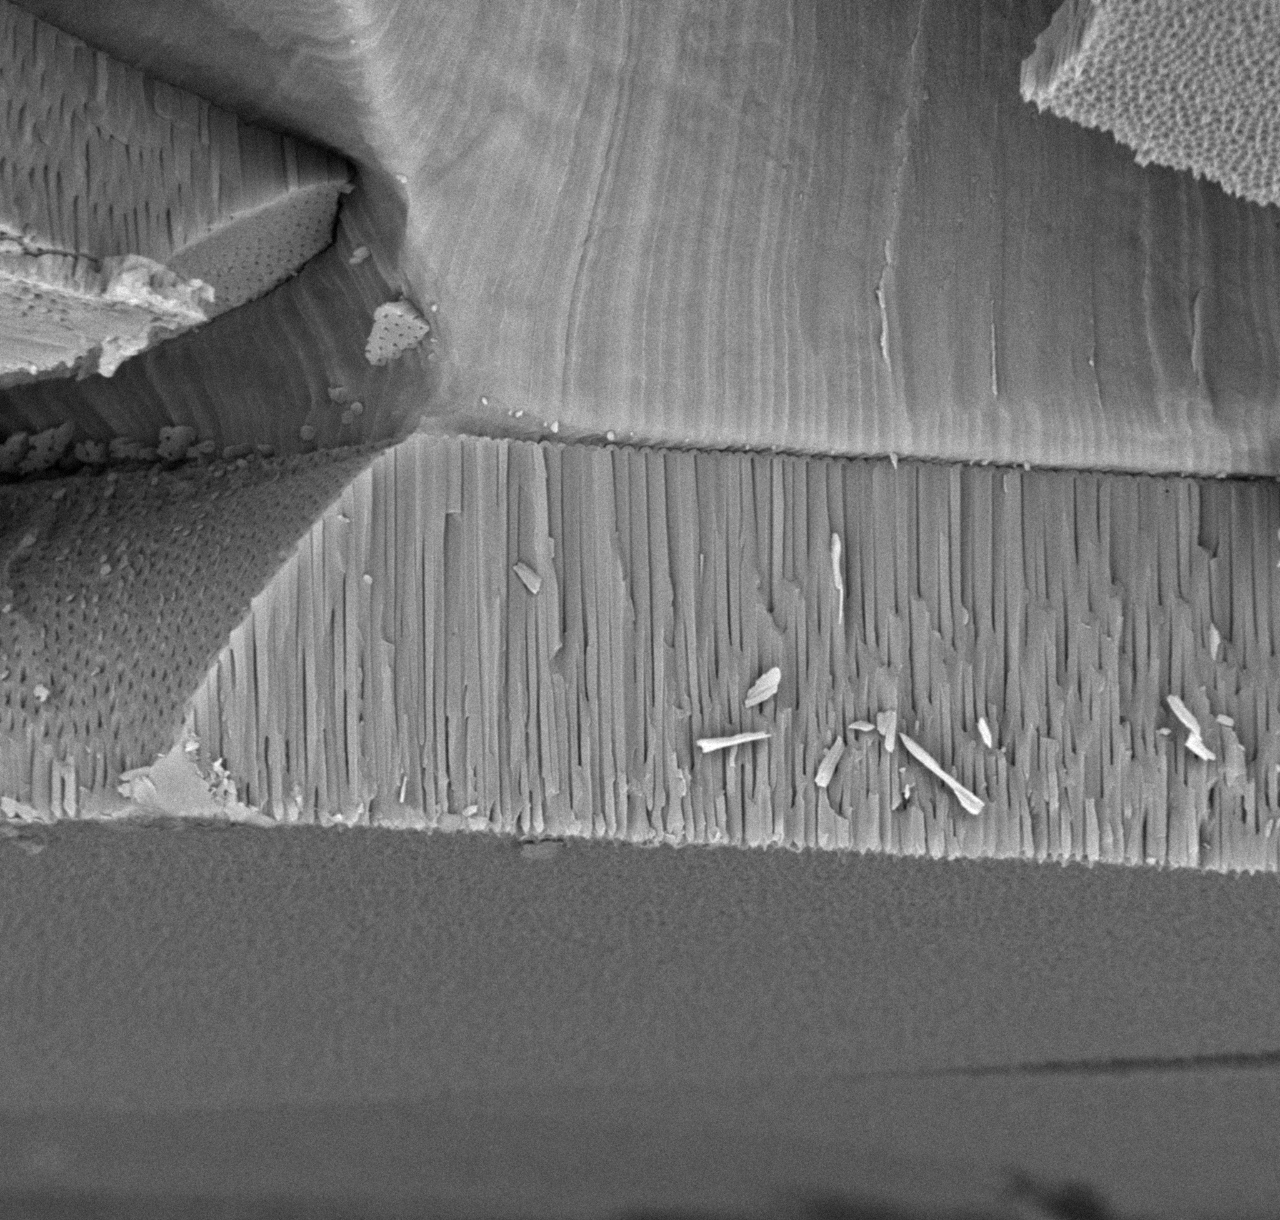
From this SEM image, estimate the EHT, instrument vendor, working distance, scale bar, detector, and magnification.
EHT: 15 kV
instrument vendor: other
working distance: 6.6 mm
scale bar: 10000 nm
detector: BSE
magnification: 5 K X